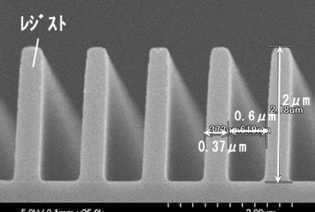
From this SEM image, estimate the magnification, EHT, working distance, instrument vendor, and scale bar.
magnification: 25 K X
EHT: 5 kV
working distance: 3.1 mm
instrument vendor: Hitachi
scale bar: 2000 nm